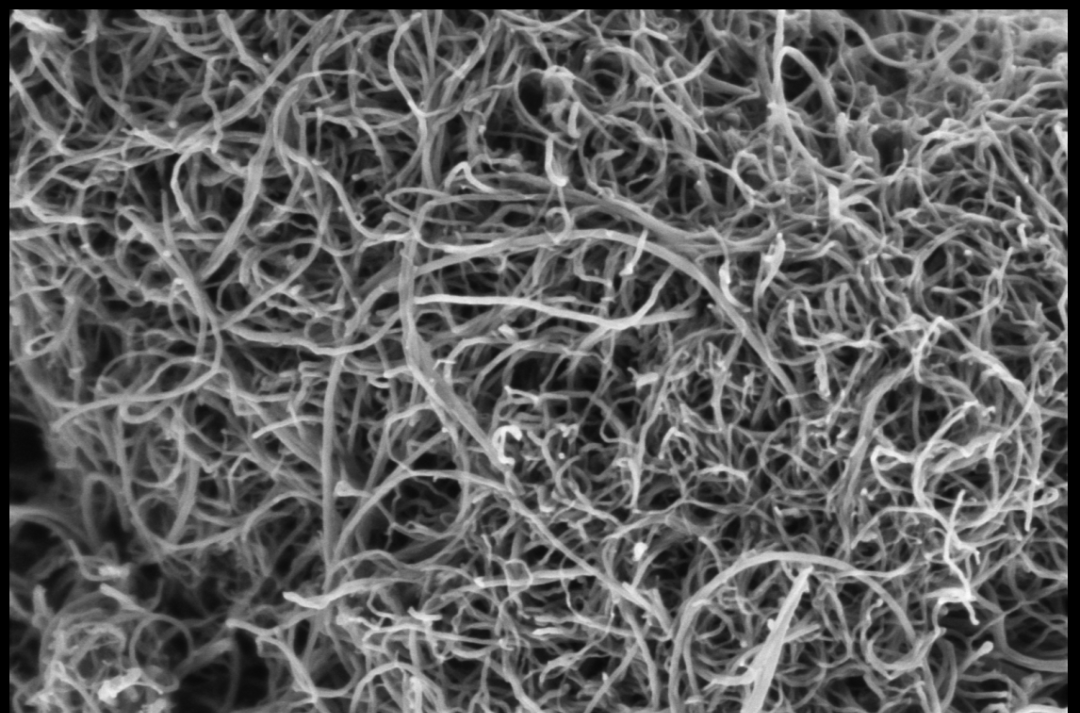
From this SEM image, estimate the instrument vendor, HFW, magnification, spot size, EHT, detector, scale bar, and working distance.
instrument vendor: Thermo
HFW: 2.54 µm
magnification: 50 K X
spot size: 6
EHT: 1 kV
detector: T2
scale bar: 500 nm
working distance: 4 mm